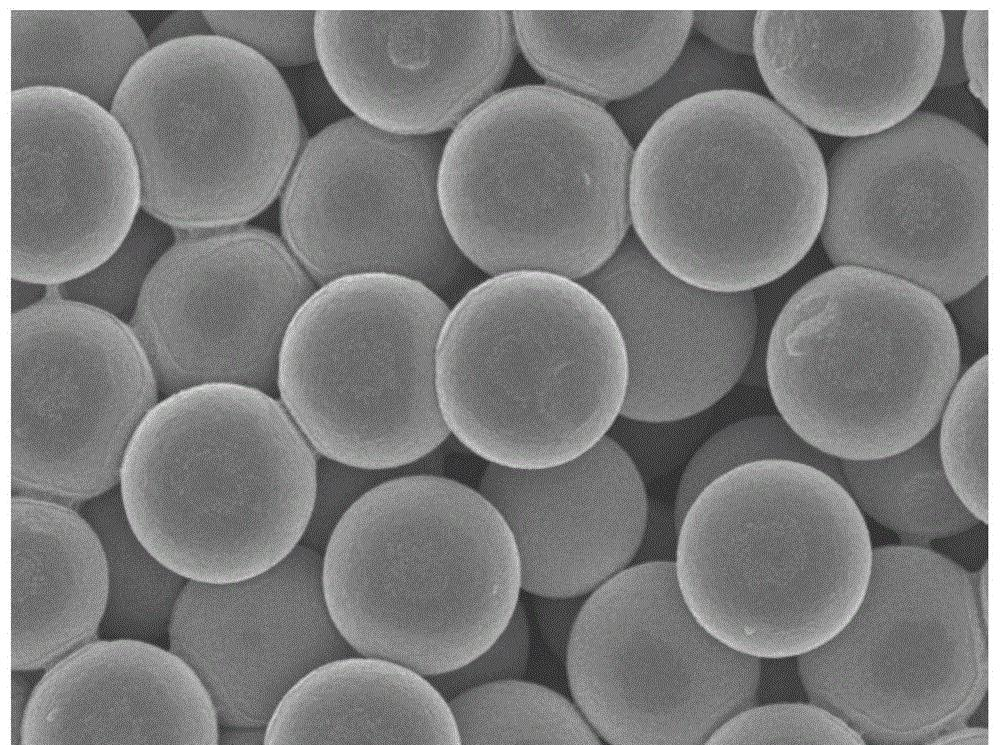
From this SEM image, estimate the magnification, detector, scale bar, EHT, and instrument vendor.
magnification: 30 K X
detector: SEI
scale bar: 100 nm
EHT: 5 kV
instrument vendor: JEOL